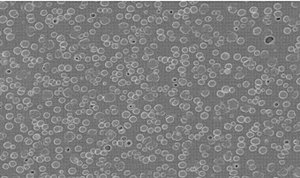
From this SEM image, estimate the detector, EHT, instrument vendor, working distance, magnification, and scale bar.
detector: InLens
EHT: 3 kV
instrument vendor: Zeiss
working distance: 4.1 mm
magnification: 5 K X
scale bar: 1000 nm